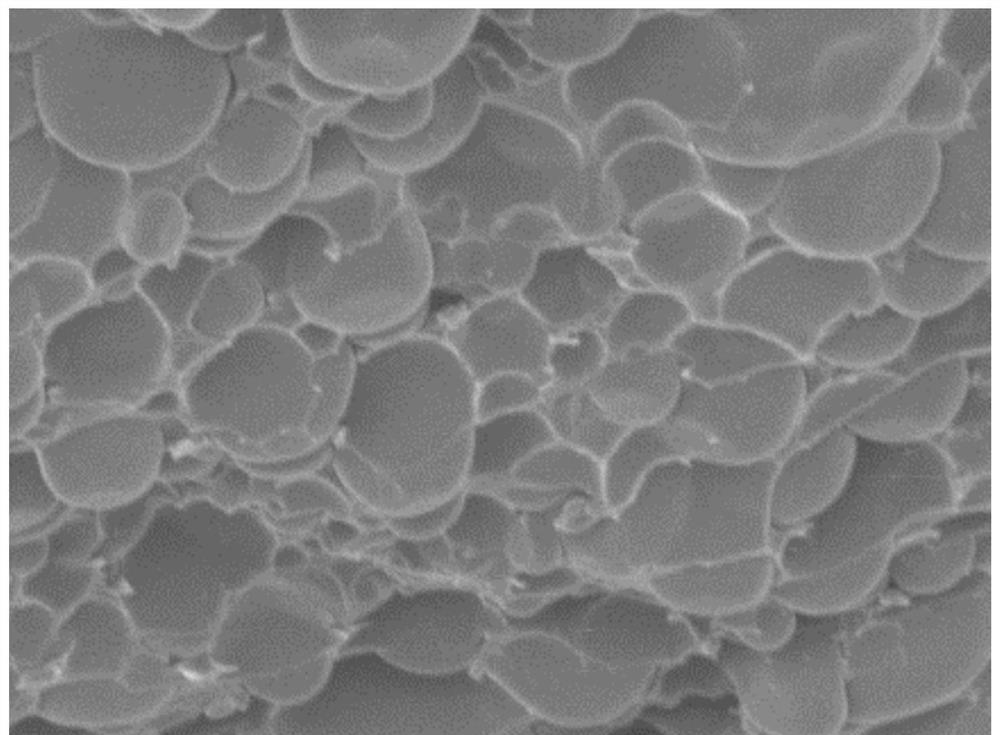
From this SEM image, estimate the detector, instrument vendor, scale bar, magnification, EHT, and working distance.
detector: SEI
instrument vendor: JEOL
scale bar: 10000 nm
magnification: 2 K X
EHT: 5 kV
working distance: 8 mm